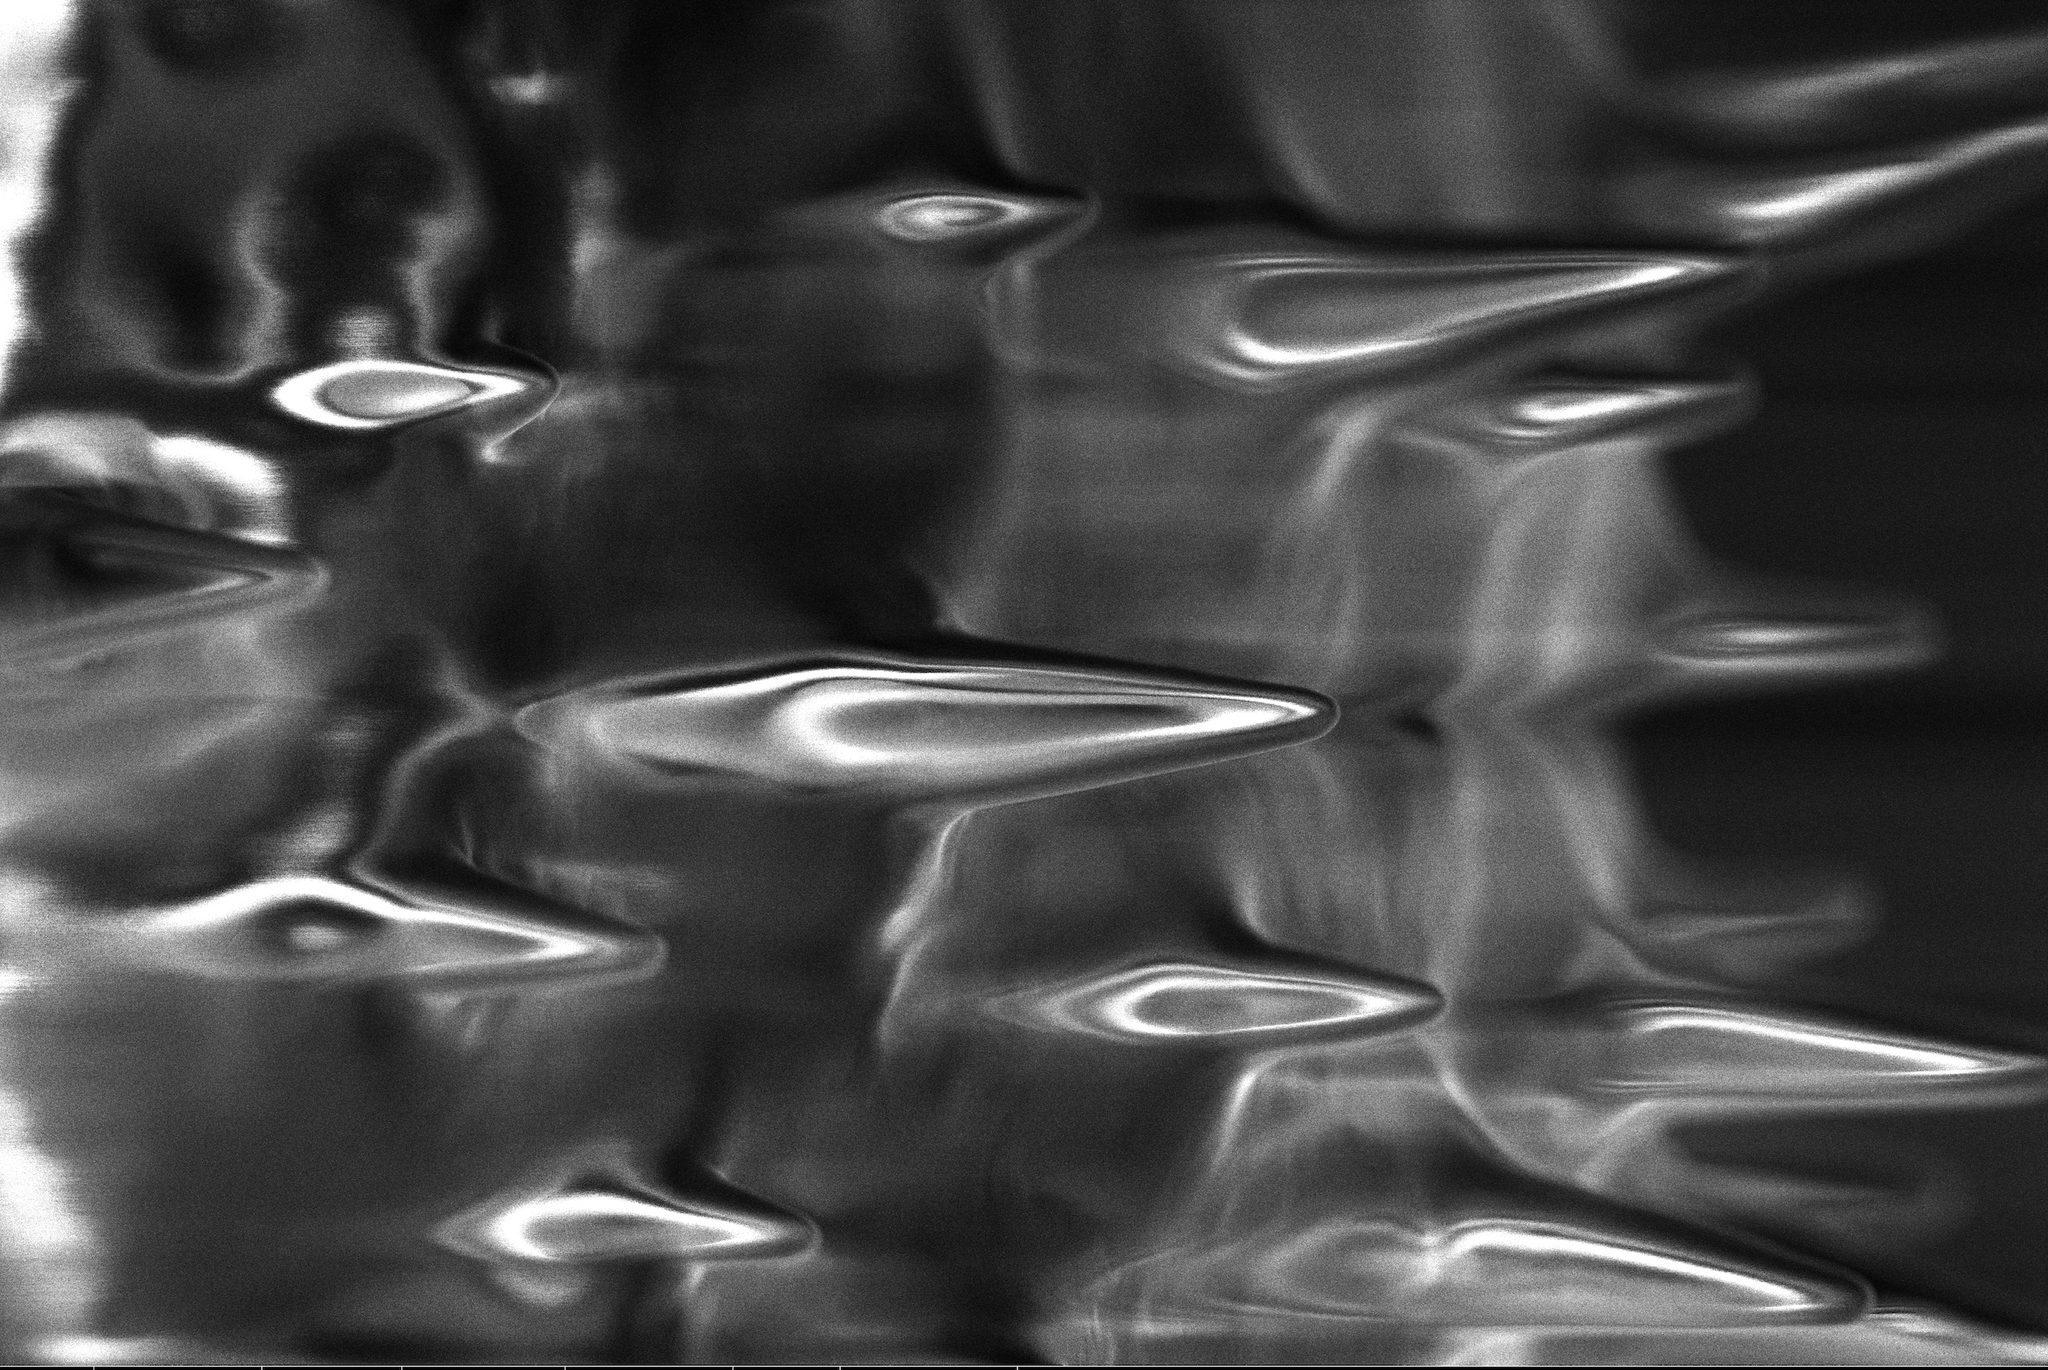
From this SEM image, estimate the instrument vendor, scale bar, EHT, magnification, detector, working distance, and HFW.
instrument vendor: FEI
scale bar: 10000 nm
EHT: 3 kV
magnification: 7.943 K X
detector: TLD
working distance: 4.5 mm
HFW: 26.1 µm